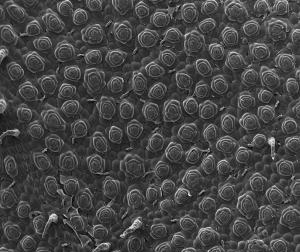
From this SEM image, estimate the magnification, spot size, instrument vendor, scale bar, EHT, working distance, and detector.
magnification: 0.1 K X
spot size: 3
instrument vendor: FEI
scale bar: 1000000 nm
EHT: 20 kV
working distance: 10.7 mm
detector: ETD